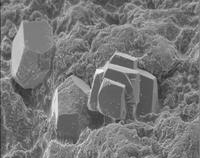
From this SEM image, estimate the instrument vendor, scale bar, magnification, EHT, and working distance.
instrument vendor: JEOL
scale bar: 10000 nm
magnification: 1 K X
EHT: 7 kV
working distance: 11 mm